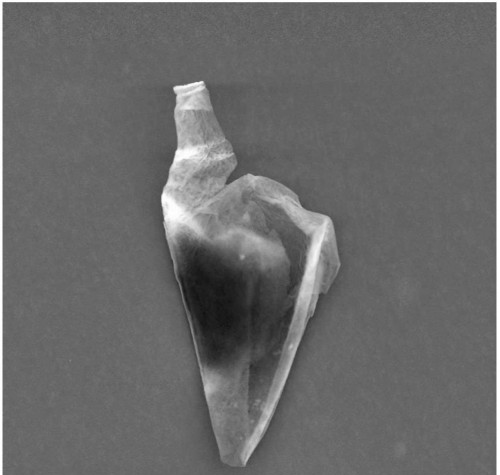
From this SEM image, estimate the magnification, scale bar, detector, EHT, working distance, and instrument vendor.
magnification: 1.9 K X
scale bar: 10000 nm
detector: SEI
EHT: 15 kV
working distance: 23.2 mm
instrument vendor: JEOL